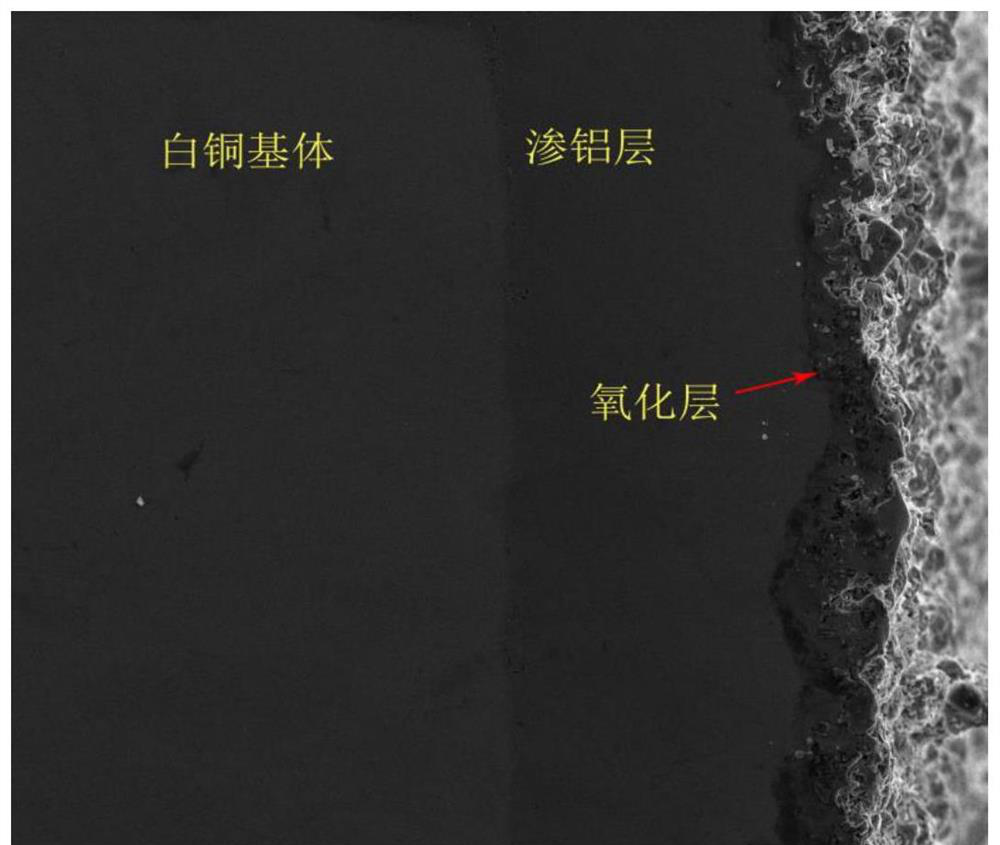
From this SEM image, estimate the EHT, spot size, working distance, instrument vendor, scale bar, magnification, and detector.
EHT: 20 kV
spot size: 3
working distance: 8 mm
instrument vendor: FEI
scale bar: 200000 nm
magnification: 0.5 K X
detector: ETD